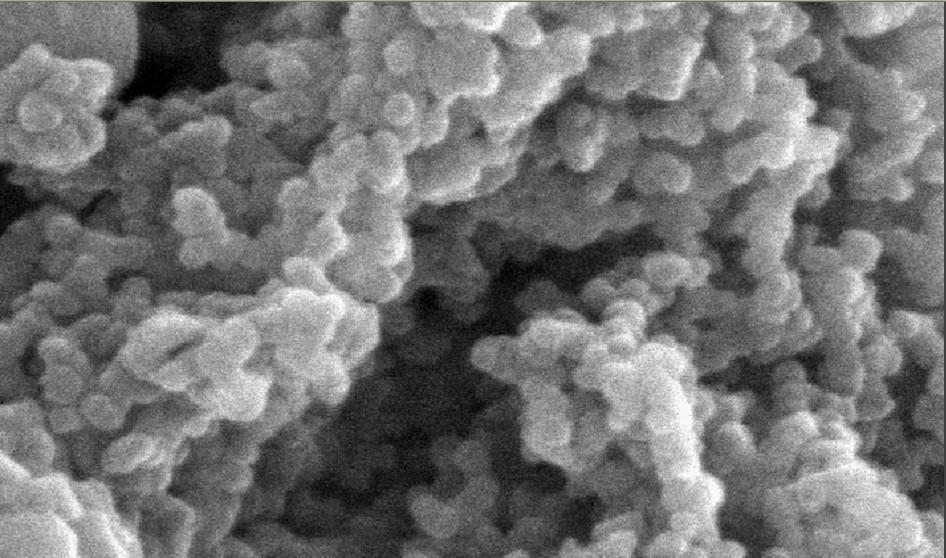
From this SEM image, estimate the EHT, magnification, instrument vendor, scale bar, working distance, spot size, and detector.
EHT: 20 kV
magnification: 80 K X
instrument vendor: FEI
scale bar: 200 nm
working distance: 14.2 mm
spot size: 2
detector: SE